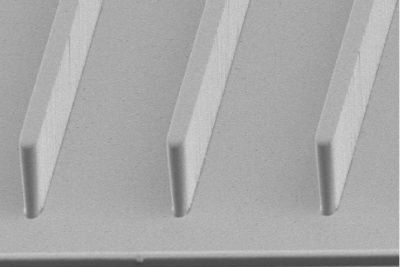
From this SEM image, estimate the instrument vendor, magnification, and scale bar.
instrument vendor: JEOL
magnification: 1 K X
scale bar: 10000 nm